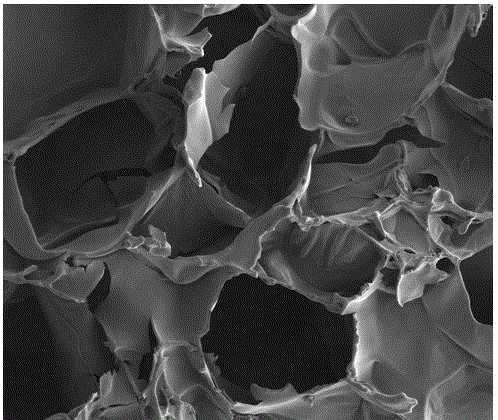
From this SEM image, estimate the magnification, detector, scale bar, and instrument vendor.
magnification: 0.5 K X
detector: ETD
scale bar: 300000 nm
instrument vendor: FEI